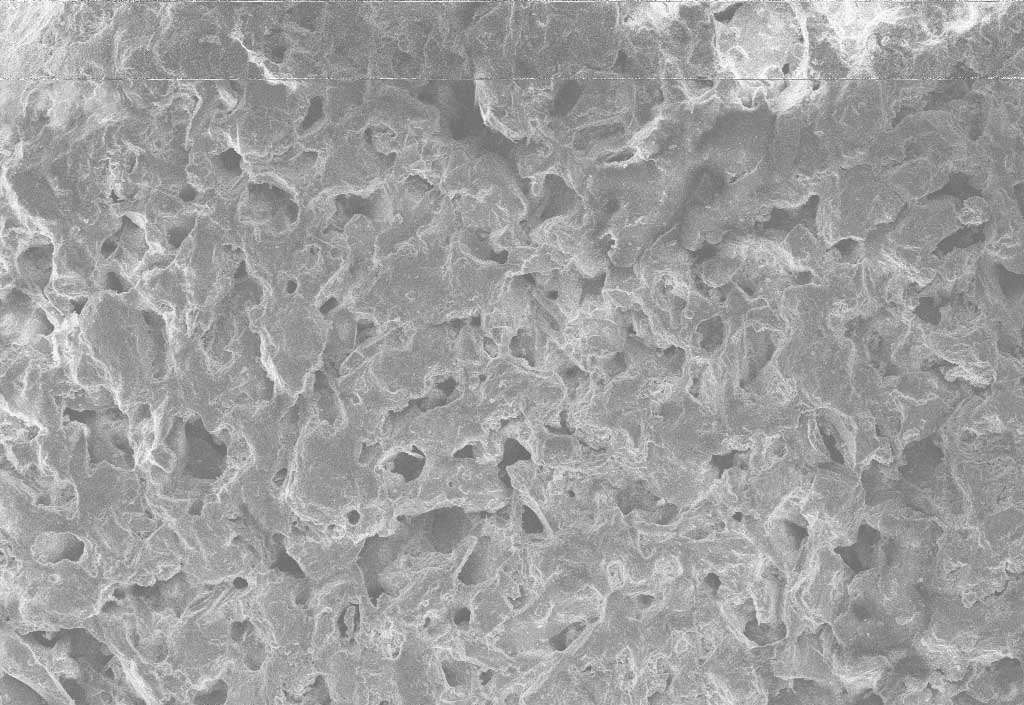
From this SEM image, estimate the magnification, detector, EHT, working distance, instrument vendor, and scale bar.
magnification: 0.051 K X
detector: SE1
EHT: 20 kV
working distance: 43 mm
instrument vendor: Zeiss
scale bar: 200000 nm